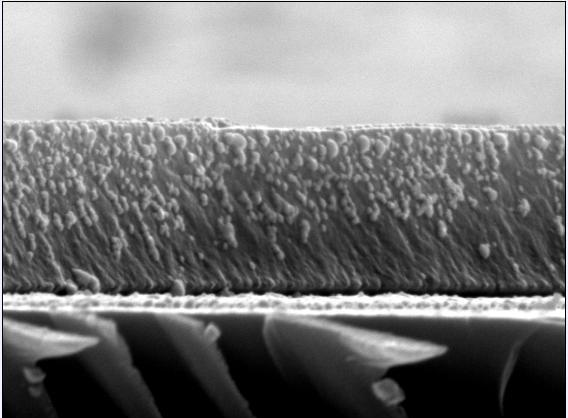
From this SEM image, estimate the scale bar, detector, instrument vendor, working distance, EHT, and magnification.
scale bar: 100 nm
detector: SEI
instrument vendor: JEOL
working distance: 9.9 mm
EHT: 10 kV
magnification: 50 K X